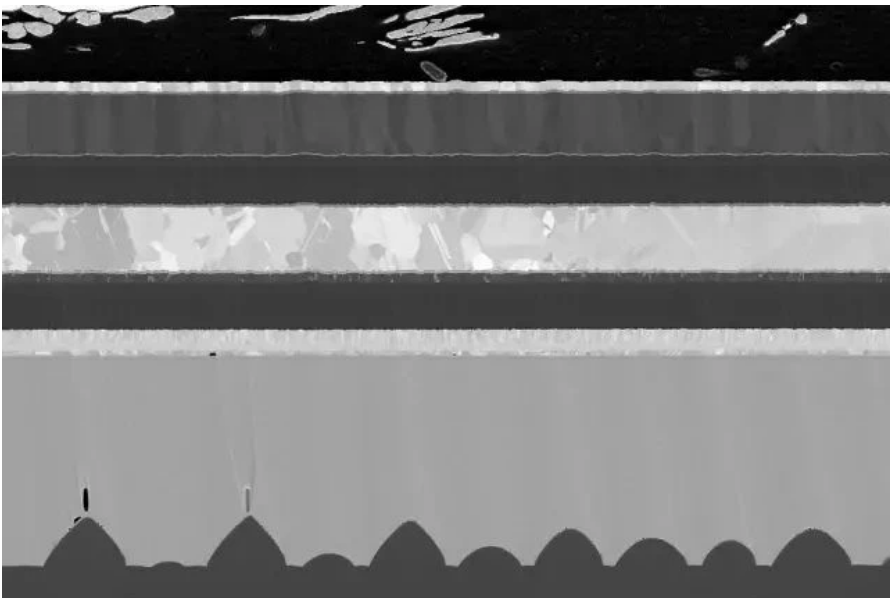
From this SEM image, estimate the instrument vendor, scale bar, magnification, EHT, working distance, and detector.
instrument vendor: Zeiss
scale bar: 1000 nm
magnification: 4 K X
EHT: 2 kV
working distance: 4.9 mm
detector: SE2